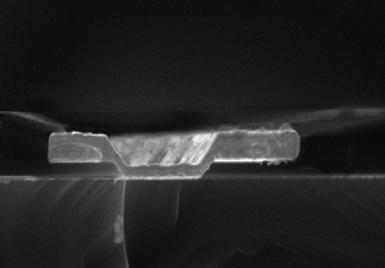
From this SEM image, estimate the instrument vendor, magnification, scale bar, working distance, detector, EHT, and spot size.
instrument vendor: FEI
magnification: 17.089 K X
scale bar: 2000 nm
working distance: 15.1 mm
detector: SE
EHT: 20 kV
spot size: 2